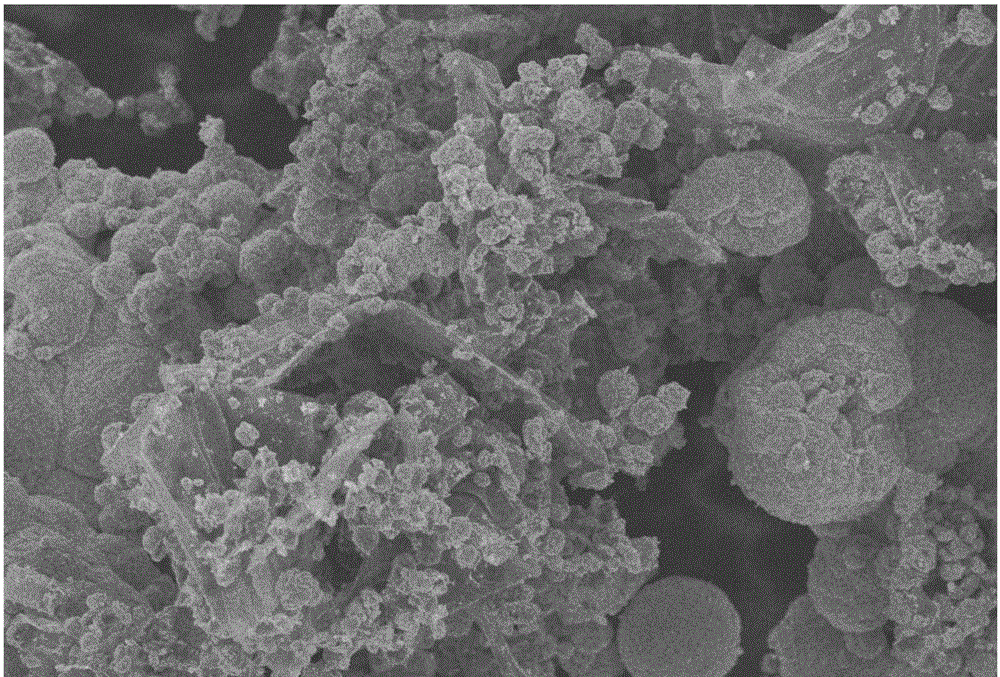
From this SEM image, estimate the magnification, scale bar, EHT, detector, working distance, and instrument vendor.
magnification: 5.1 K X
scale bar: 1000 nm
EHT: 3 kV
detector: InLens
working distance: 5.8 mm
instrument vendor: Zeiss